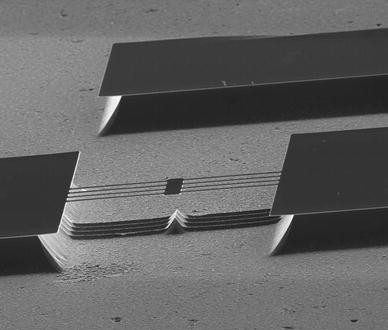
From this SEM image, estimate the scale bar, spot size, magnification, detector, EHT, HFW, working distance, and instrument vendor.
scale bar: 100000 nm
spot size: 3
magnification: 0.6 K X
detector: ETD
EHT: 10 kV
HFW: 400 µm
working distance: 11.4 mm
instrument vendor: FEI